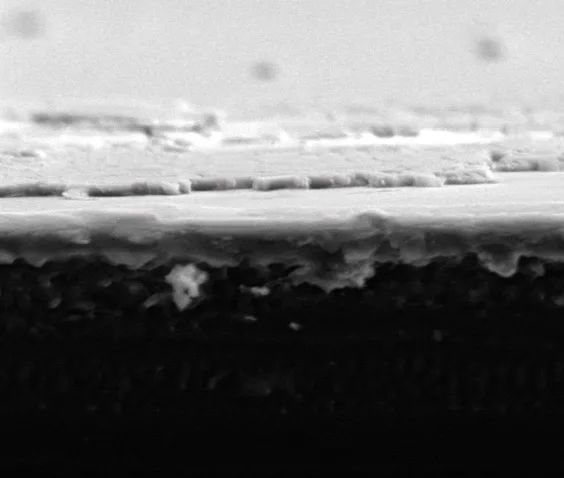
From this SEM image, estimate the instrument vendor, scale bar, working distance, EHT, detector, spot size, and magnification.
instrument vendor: FEI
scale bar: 20000 nm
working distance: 12.9 mm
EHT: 10 kV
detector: LFD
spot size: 4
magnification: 5 K X